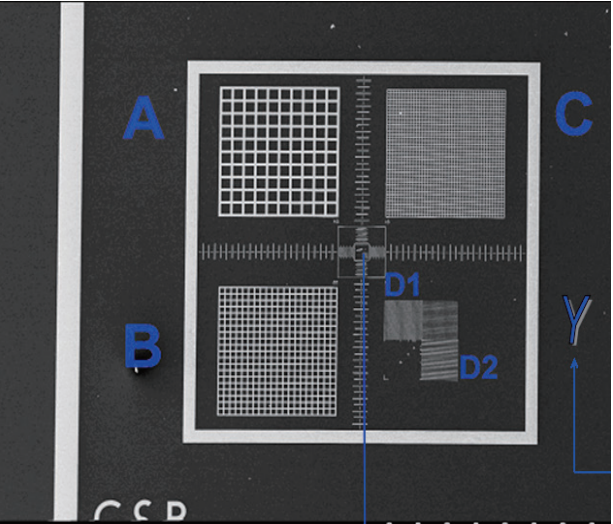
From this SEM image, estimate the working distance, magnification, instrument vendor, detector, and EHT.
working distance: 13.1 mm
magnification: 0.05 K X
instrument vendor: JEOL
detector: SE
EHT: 10 kV